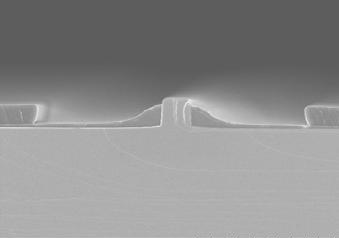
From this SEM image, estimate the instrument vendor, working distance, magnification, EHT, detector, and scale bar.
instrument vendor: Hitachi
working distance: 12.2 mm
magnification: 5 K X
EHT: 5 kV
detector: SE(U)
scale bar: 10000 nm